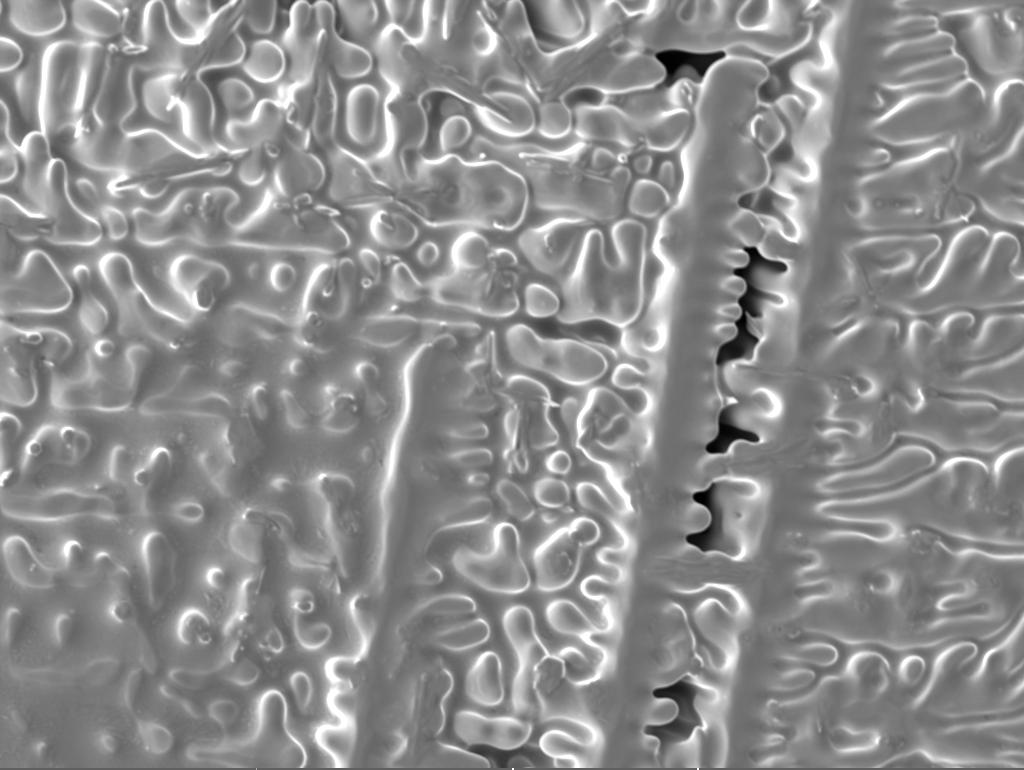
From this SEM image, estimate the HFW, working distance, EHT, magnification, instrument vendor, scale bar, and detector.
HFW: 277 µm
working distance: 12.13 mm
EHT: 20 kV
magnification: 1 K X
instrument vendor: Tescan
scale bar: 50000 nm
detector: SE